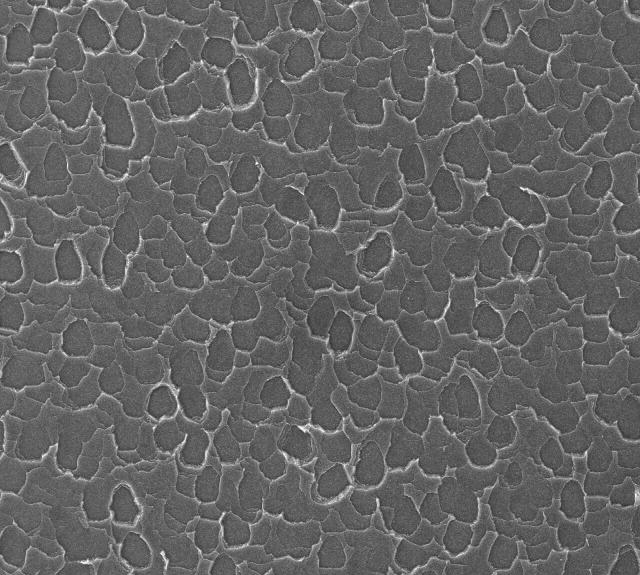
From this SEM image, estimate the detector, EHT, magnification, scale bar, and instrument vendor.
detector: SEI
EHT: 30 kV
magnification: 0.16 K X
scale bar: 100000 nm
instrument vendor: JEOL